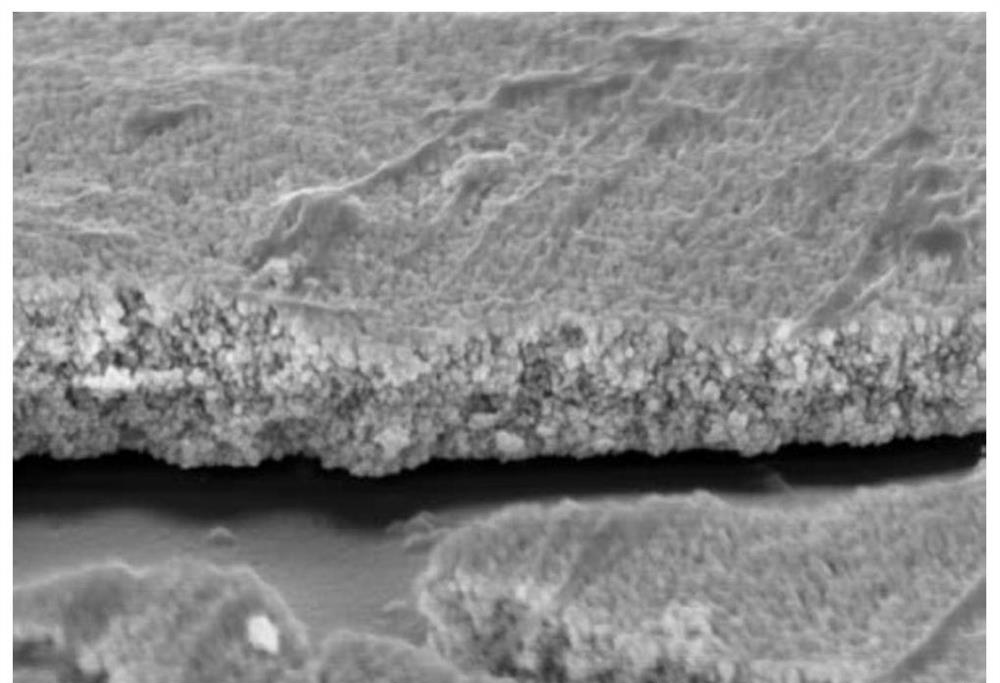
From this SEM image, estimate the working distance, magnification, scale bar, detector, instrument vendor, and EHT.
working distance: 9.2 mm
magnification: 40 K X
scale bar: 1000 nm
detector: SE(UL)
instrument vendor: Hitachi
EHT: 5 kV